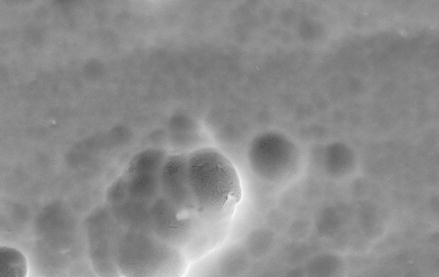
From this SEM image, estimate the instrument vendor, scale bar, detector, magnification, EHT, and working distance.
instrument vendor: Zeiss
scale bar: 2000 nm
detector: SE1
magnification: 10 K X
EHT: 20 kV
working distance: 10.5 mm